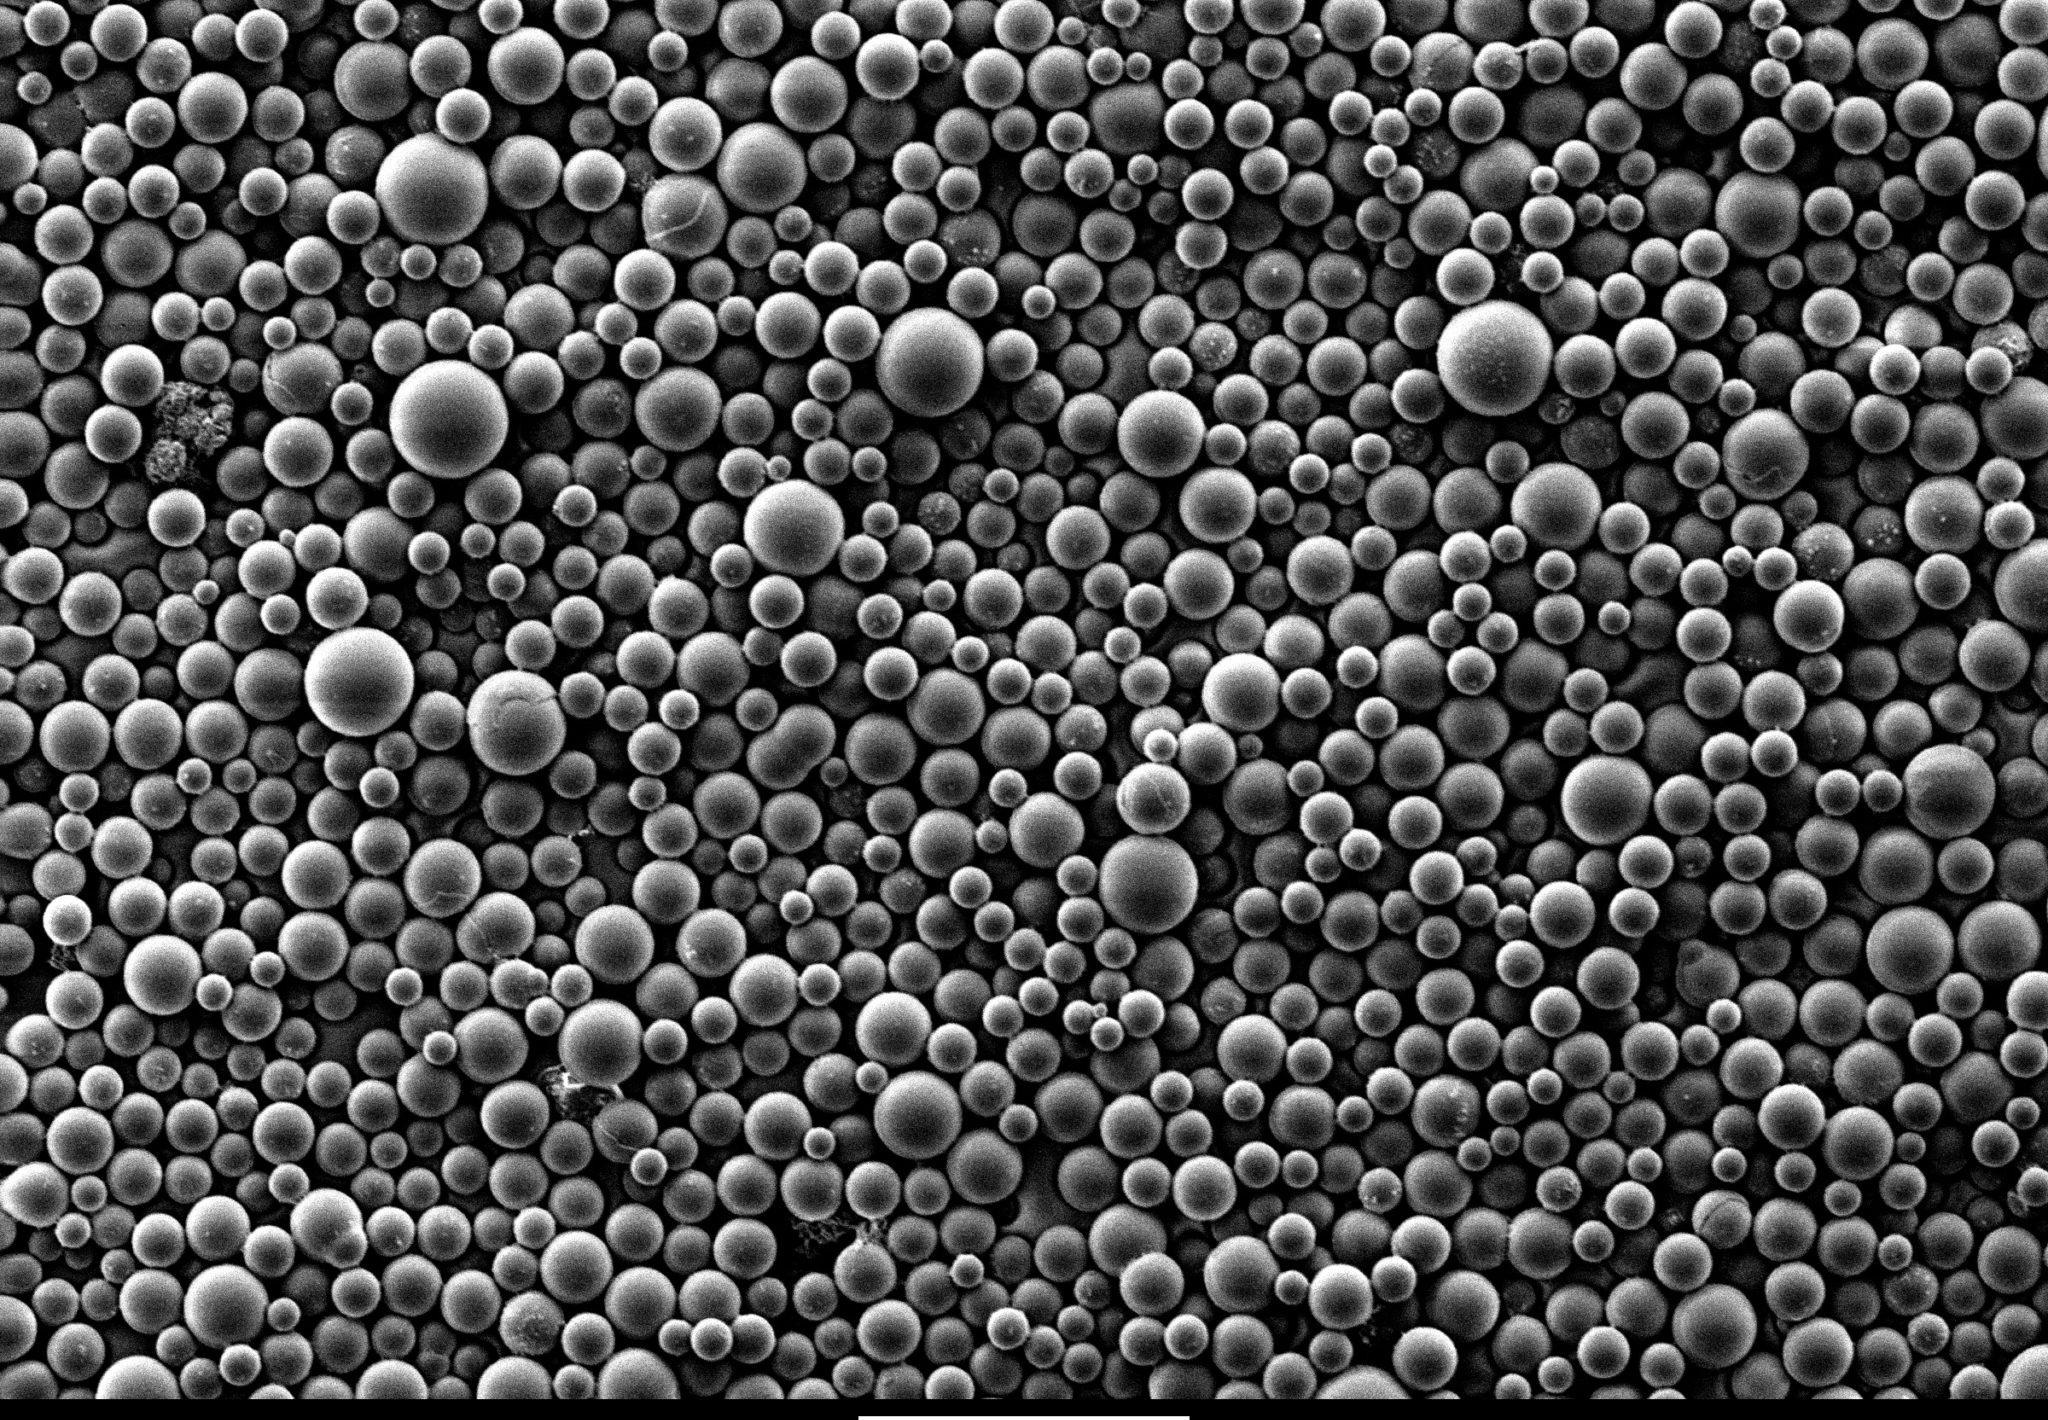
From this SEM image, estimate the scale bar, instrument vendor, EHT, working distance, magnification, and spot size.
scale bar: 100000 nm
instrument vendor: FEI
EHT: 5 kV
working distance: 8 mm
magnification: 0.196 K X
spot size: -1